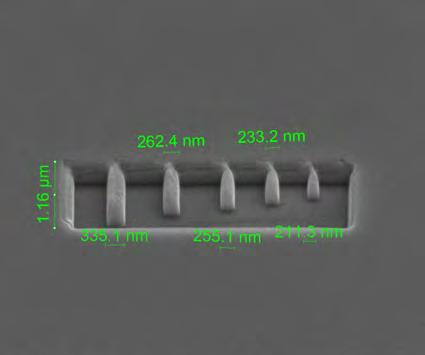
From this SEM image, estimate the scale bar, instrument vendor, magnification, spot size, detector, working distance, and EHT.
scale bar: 2000 nm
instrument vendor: FEI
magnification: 20 K X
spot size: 3.5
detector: ETD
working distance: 10 mm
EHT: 5 kV